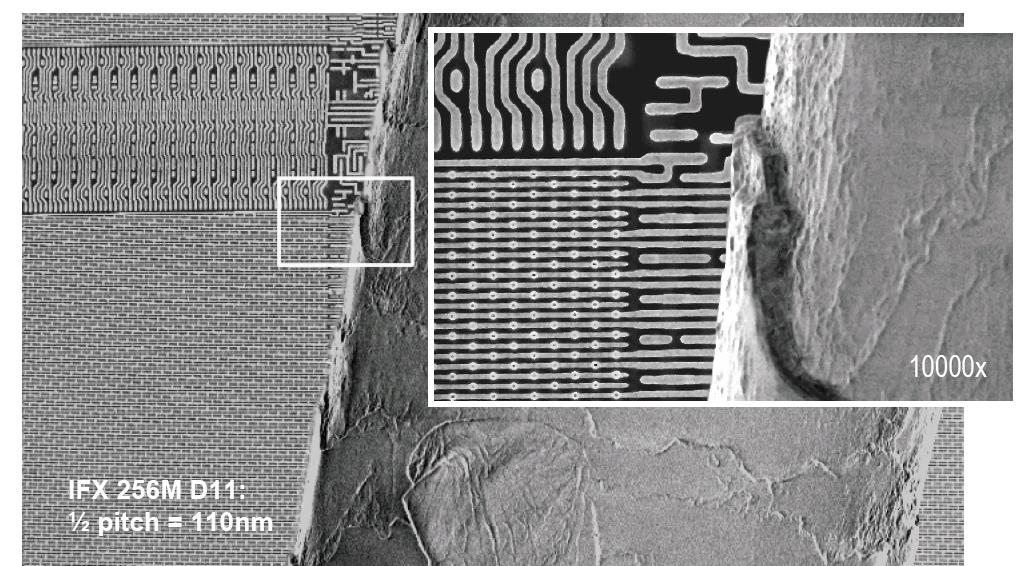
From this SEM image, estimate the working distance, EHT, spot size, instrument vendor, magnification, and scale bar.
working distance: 5 mm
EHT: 10 kV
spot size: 2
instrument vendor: FEI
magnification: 1.5 K X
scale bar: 20000 nm